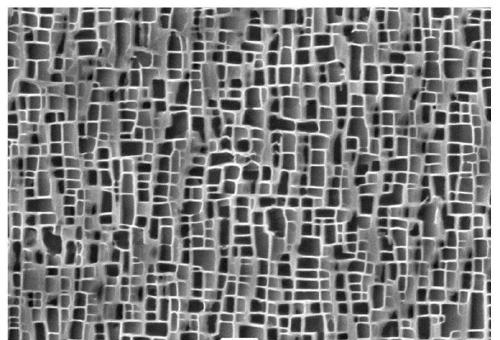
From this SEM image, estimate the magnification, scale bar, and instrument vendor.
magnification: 10 K X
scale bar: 1000 nm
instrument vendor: JEOL